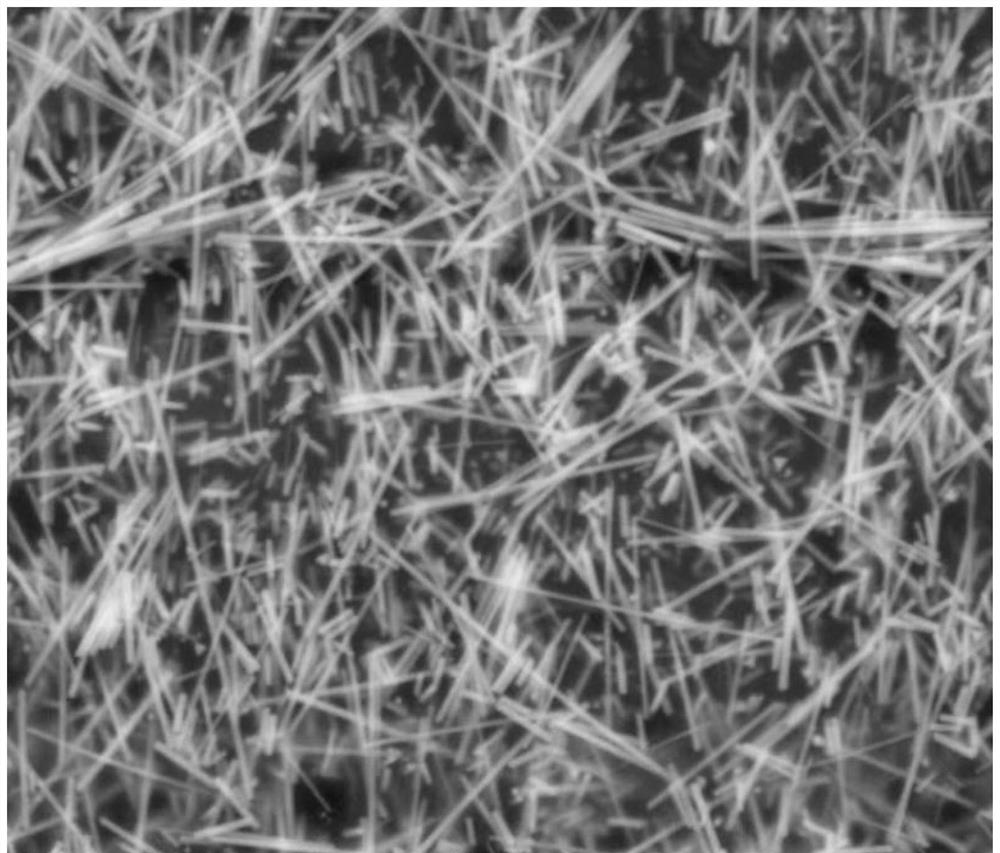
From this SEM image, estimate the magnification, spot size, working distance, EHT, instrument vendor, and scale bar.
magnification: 10 K X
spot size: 4.5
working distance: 9.6 mm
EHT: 30 kV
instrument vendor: FEI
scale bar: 10000 nm